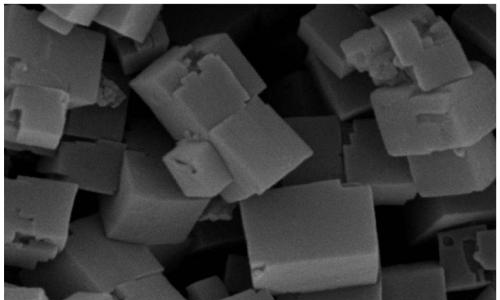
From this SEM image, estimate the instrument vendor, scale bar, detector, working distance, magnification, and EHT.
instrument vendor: Hitachi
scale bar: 1000 nm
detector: SE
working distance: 5 mm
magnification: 30 K X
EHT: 15 kV